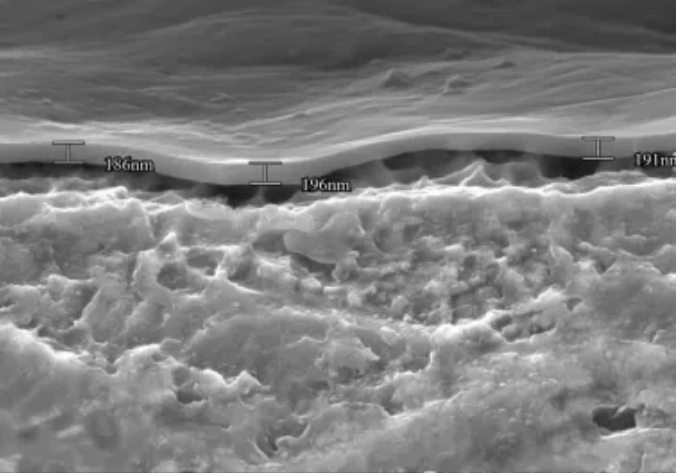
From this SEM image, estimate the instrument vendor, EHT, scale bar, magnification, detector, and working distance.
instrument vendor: Hitachi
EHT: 15 kV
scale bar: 2000 nm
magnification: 20 K X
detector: SE(U)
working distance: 14.8 mm